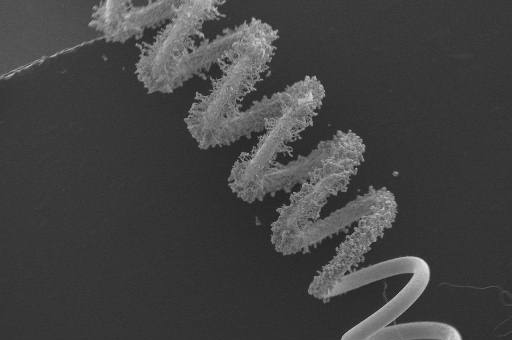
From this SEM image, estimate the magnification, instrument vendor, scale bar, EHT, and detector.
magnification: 0.022 K X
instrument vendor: JEOL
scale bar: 1000000 nm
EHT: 15 kV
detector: SEI